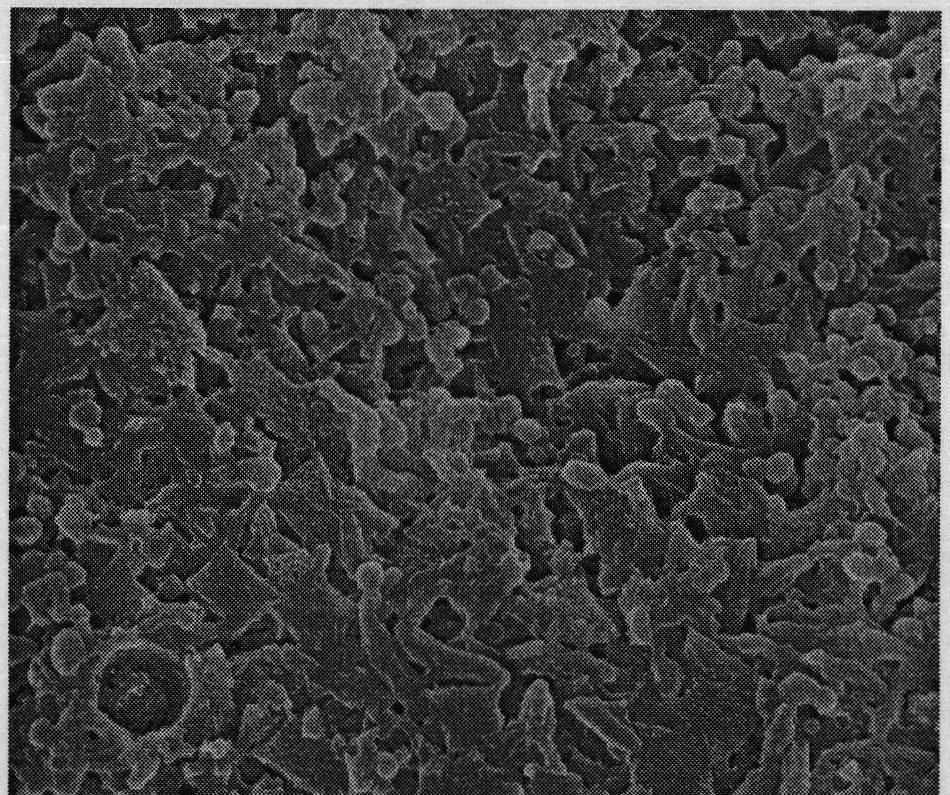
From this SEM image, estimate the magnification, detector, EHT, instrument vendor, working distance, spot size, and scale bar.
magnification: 10 K X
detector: ETD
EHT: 25 kV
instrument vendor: FEI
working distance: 10.8 mm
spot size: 3.5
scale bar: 5000 nm